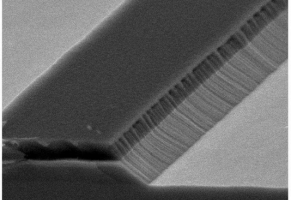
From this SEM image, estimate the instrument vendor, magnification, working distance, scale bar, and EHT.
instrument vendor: JEOL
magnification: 30 K X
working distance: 18 mm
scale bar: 1000 nm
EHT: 5 kV